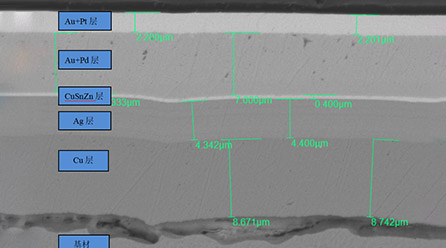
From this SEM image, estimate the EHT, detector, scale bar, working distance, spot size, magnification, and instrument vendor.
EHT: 10 kV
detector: BEC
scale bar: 5000 nm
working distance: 10 mm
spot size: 40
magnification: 3 K X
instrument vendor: JEOL